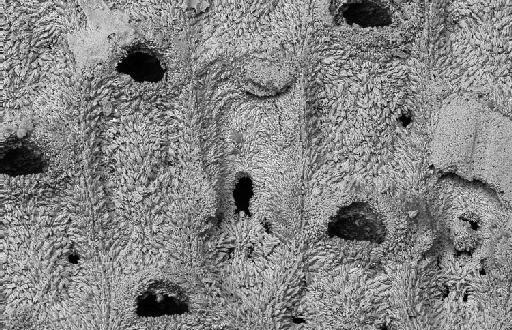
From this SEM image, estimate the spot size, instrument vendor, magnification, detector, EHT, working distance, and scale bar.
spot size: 500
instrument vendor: Zeiss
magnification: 0.35 K X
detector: QBSD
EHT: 20 kV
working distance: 16 mm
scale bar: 100000 nm